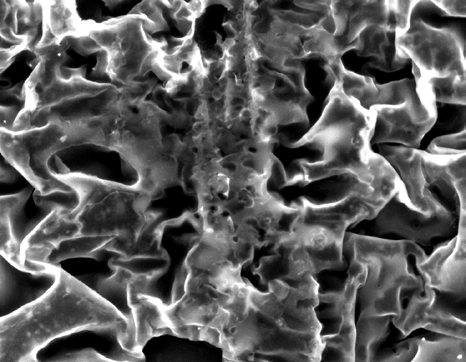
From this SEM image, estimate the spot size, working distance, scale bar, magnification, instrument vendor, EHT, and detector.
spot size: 5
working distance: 12 mm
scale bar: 50000 nm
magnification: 1 K X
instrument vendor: FEI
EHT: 30 kV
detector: ETD